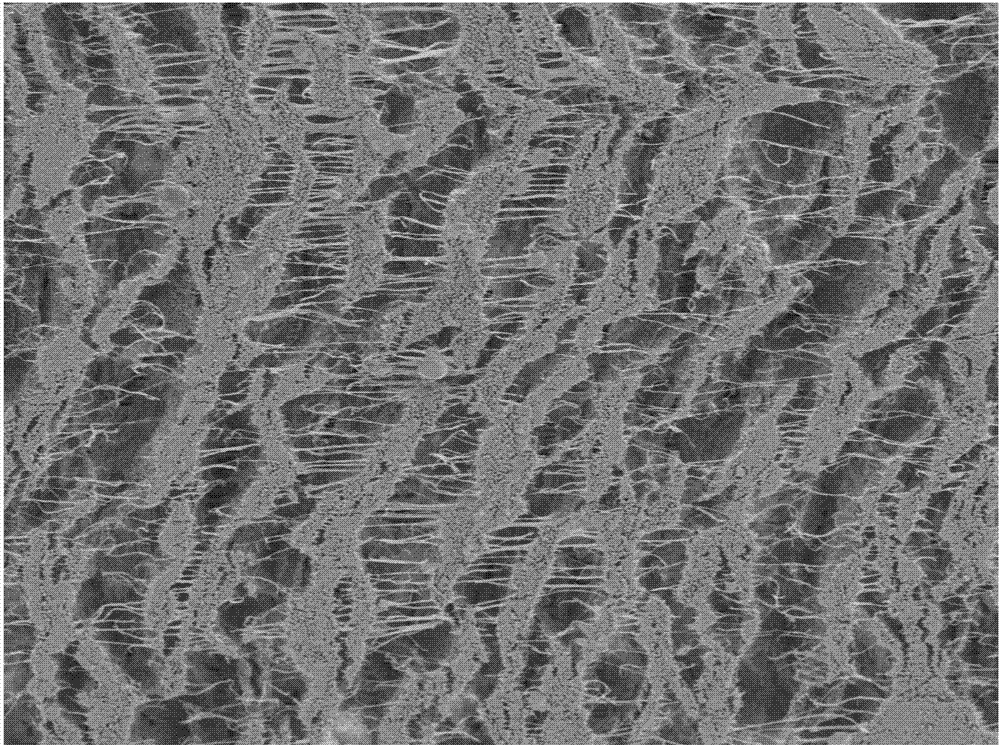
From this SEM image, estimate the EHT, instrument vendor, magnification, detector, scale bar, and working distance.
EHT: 3 kV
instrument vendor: JEOL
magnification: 0.5 K X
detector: LED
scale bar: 10000 nm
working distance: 15 mm